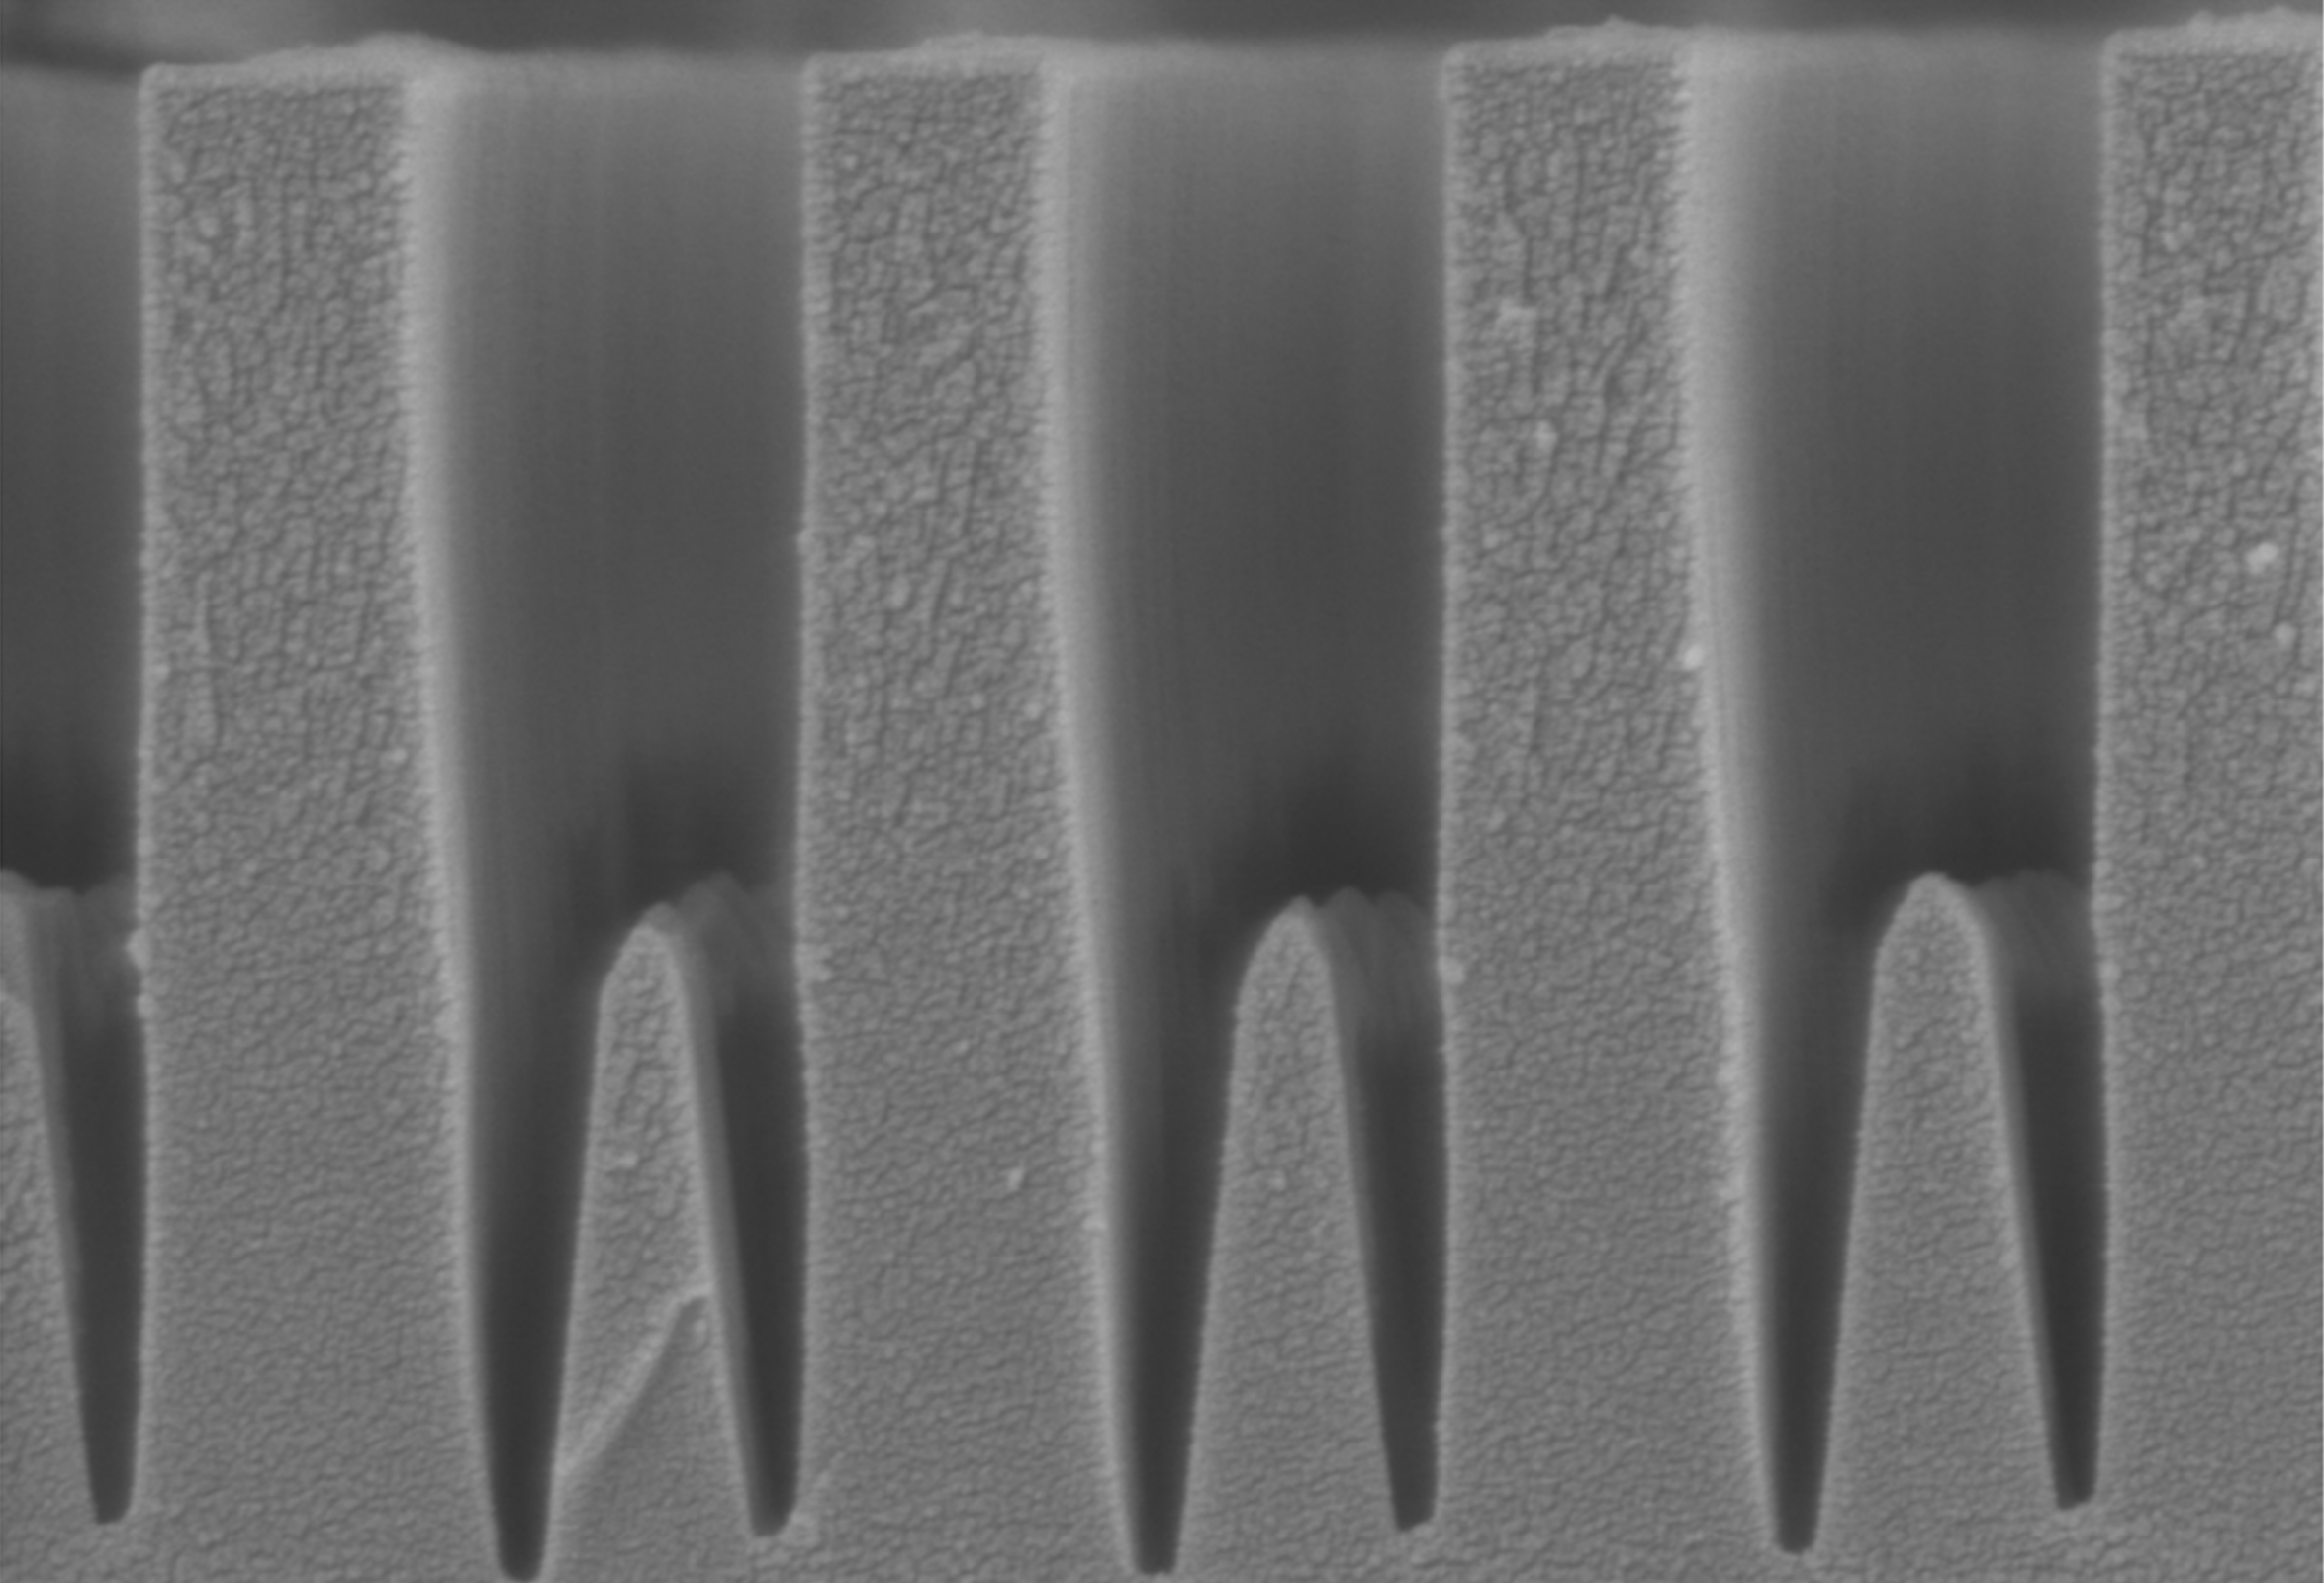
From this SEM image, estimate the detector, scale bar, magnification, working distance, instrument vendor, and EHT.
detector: SE(UL)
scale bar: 1000 nm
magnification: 50 K X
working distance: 4.7 mm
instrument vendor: Hitachi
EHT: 1.5 kV